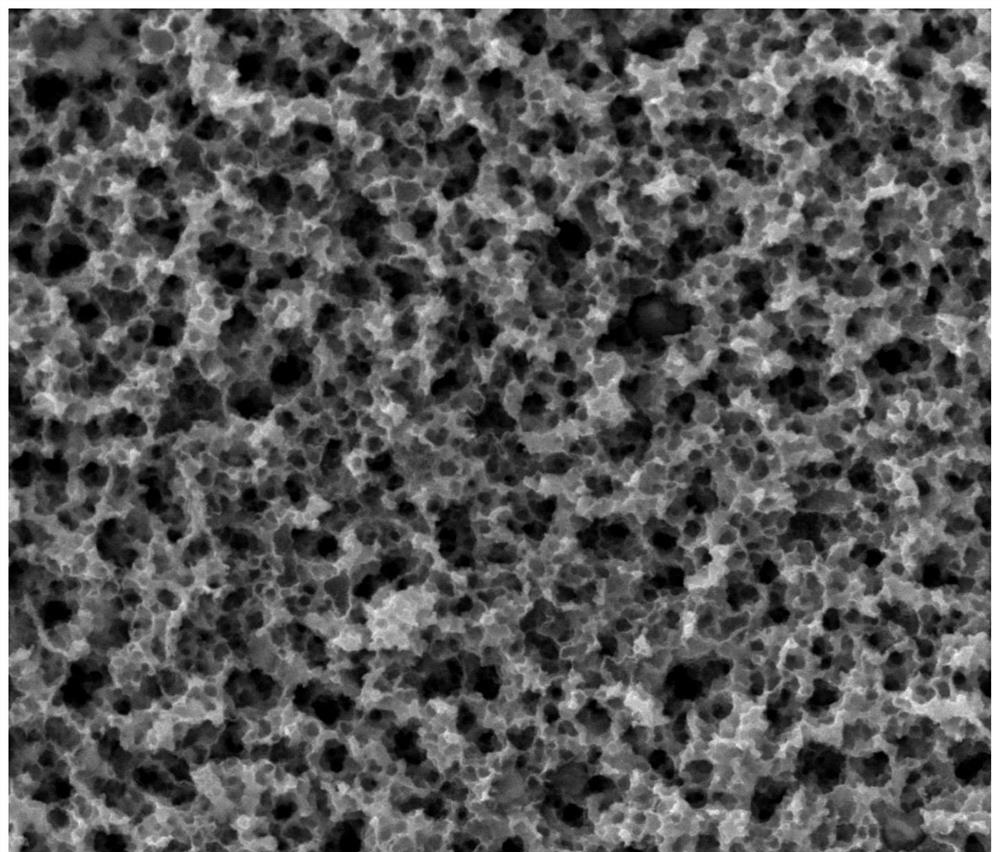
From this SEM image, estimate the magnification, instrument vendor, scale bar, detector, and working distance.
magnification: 20 K X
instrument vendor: FEI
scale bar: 5000 nm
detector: SE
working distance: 9.8 mm